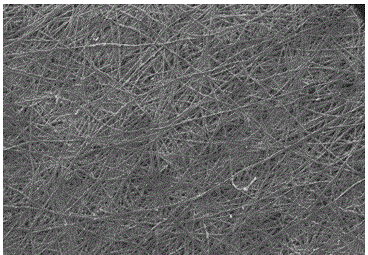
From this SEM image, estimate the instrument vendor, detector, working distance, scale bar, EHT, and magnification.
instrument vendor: Hitachi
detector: LM(L)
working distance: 11.4 mm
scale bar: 1000000 nm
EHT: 15 kV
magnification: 0.03 K X